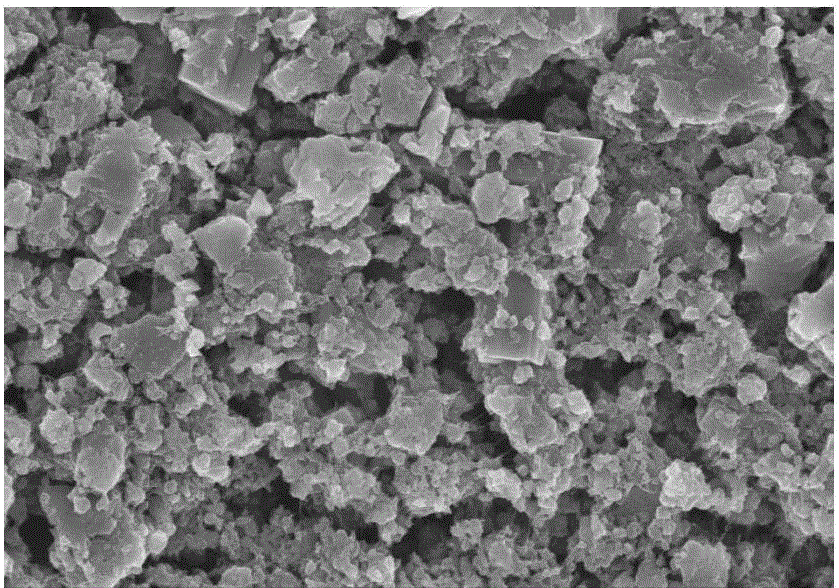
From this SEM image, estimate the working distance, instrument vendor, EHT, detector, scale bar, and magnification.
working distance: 8.4 mm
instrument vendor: Hitachi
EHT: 10 kV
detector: SE(M)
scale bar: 10000 nm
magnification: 5 K X